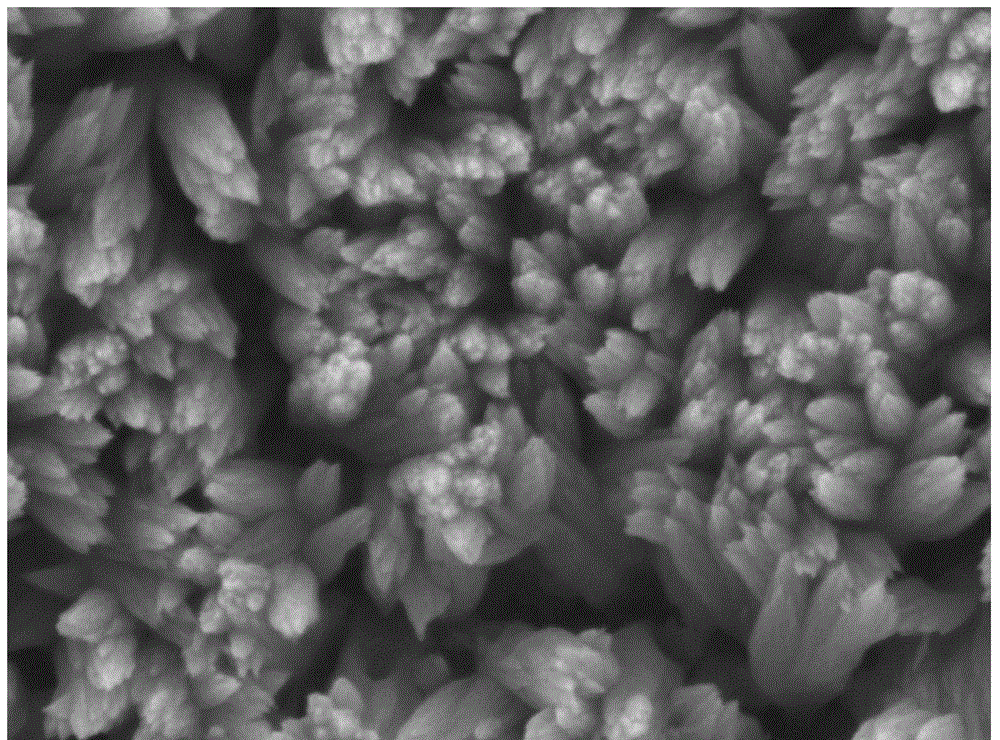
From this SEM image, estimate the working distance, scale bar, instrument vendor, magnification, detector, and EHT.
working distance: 10.1 mm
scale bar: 100 nm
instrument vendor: JEOL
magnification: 50 K X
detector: LED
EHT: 5 kV